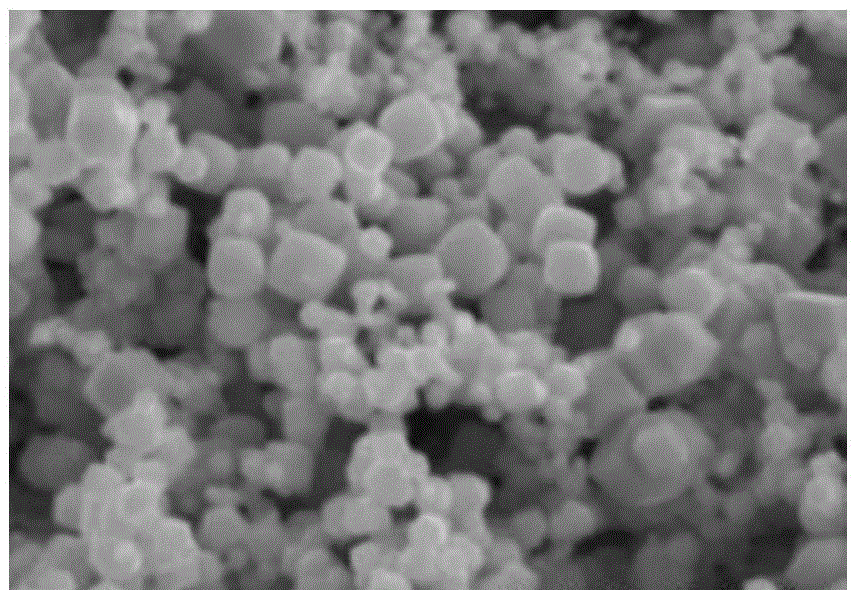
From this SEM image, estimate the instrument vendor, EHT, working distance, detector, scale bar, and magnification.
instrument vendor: Hitachi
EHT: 20 kV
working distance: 15.8 mm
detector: SE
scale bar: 5000 nm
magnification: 10 K X